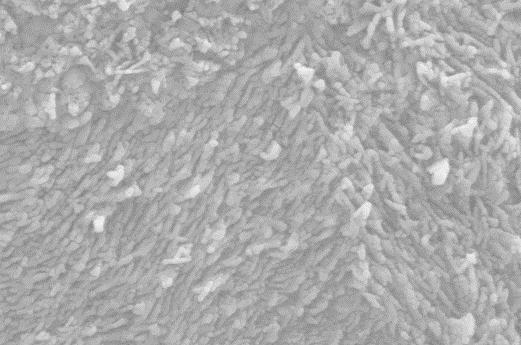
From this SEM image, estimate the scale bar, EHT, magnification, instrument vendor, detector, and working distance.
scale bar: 200 nm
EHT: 10 kV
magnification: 100.71 K X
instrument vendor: Zeiss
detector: SE2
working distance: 7.1 mm